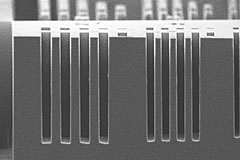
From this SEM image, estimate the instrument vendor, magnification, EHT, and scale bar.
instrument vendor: Hitachi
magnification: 1 K X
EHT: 10 kV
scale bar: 50000 nm